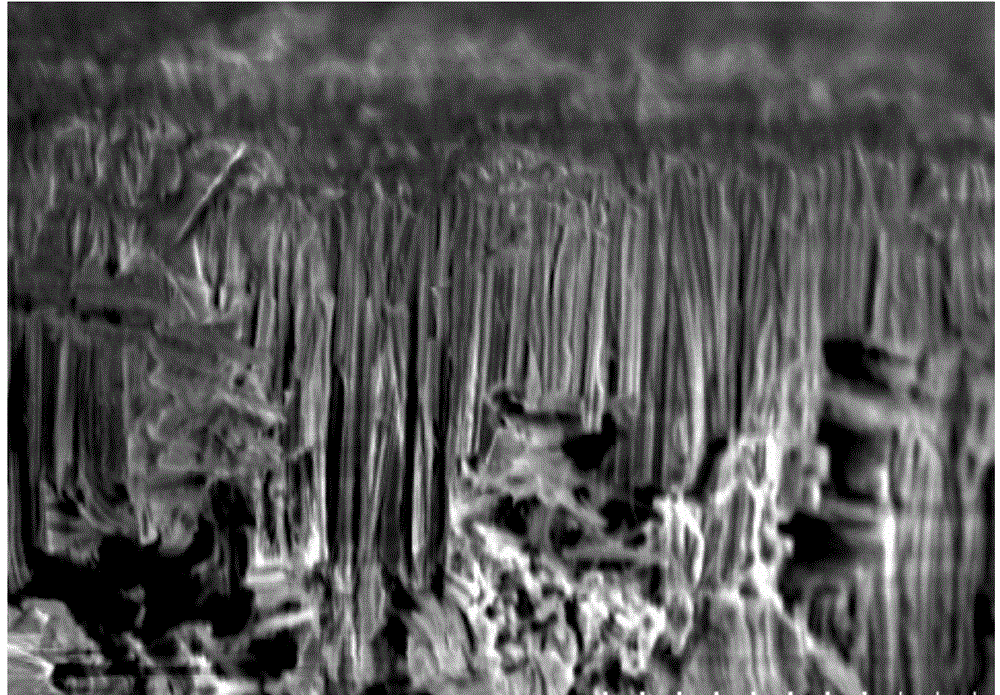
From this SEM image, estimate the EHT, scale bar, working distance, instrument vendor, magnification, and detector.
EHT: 5 kV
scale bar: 50000 nm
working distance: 11.1 mm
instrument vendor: Hitachi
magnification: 0.95 K X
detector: SE